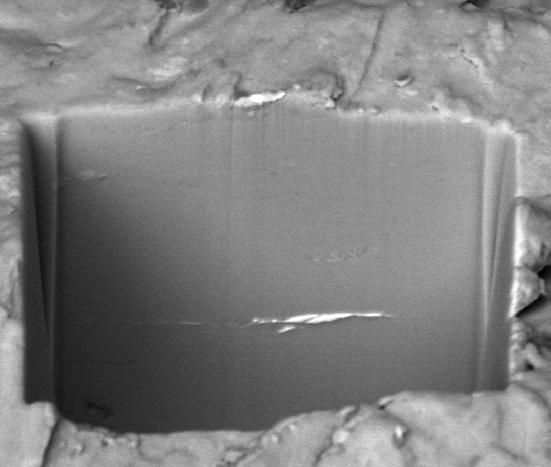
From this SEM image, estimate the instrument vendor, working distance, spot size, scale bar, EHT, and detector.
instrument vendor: FEI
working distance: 10 mm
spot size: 7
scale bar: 5000 nm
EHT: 10 kV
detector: BSED